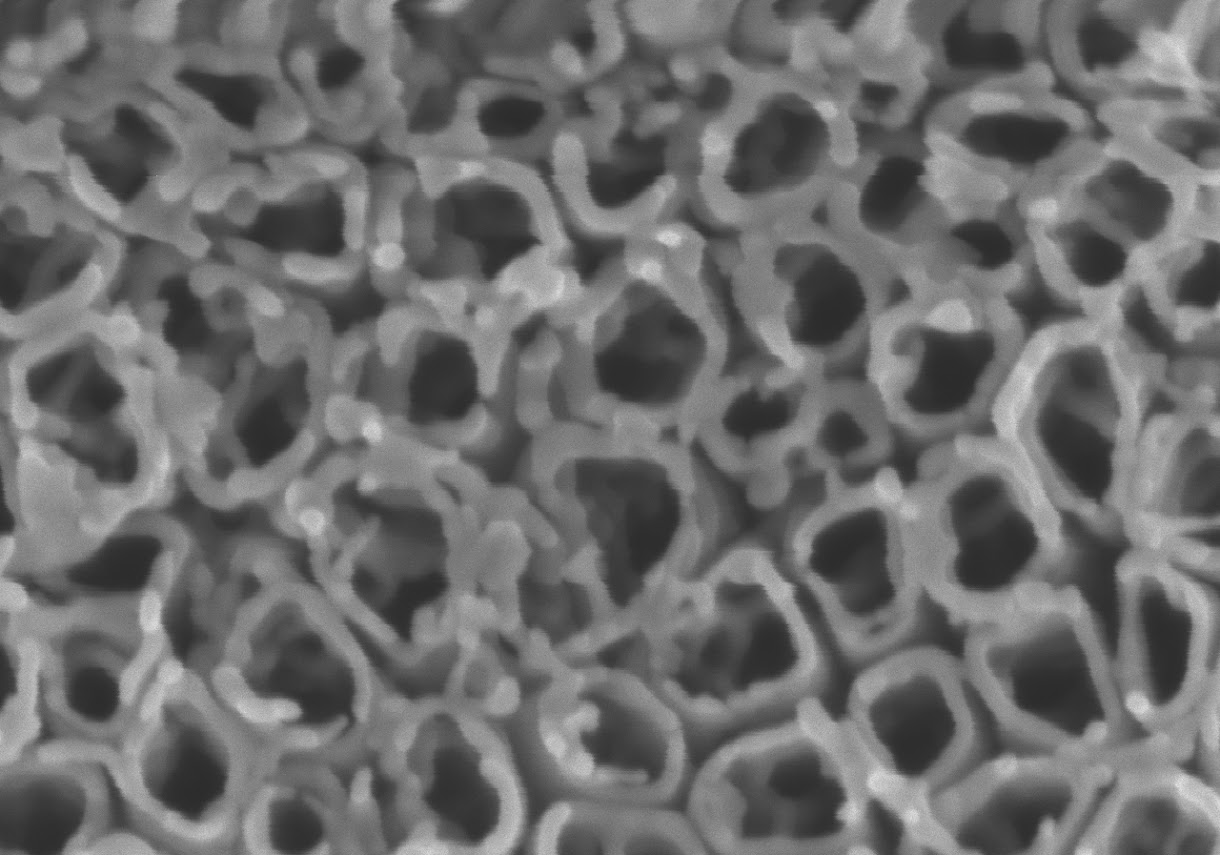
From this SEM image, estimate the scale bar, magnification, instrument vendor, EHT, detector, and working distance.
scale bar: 500 nm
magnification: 100 K X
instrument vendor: Hitachi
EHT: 2 kV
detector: SE(U)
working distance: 7.8 mm